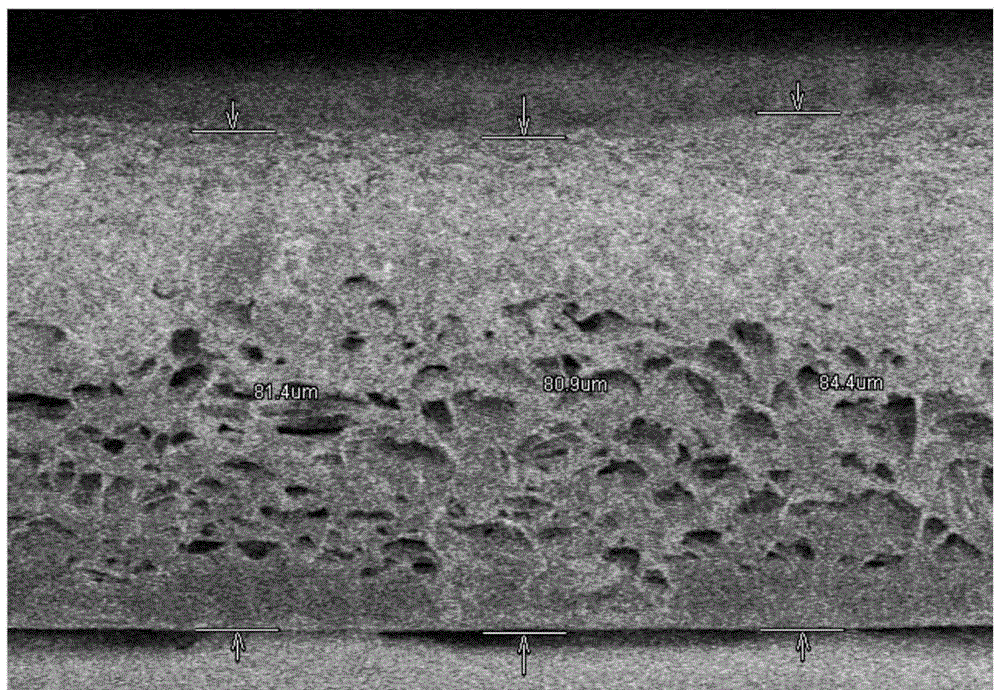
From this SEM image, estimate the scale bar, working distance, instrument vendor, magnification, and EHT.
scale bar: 50000 nm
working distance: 8.8 mm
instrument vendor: Hitachi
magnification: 0.79 K X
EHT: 3 kV